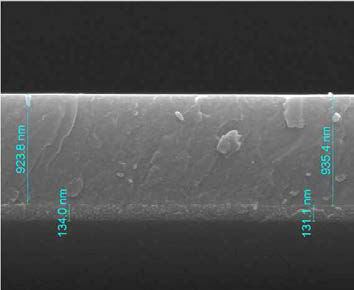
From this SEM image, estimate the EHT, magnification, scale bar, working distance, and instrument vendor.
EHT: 20 kV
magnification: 50 K X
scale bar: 1000 nm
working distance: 9.7 mm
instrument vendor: FEI